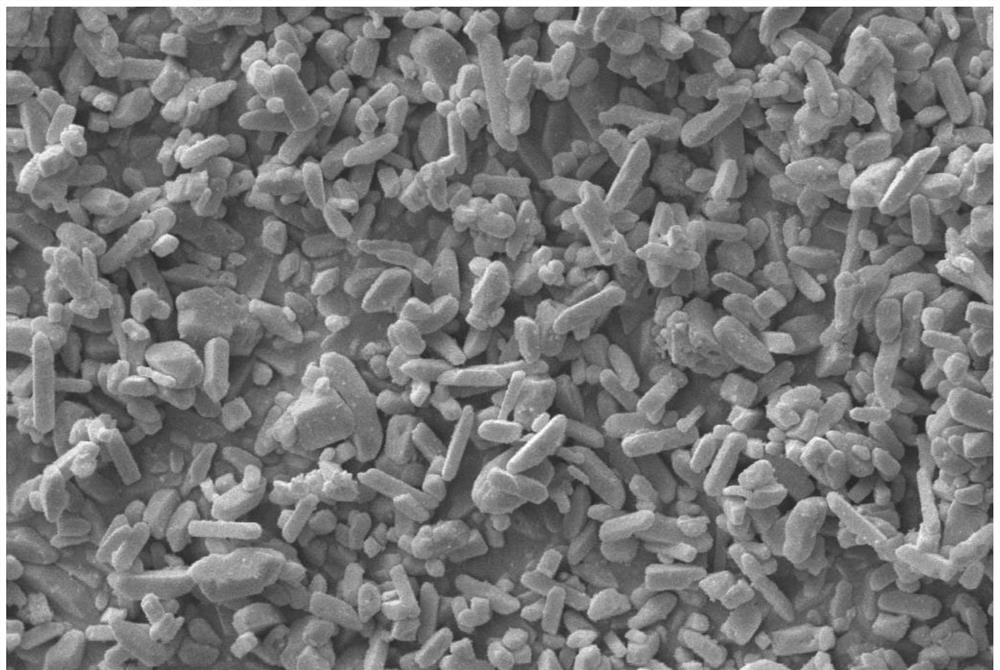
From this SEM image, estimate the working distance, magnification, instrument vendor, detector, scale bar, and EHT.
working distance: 8.5 mm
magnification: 5 K X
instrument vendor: Zeiss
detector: SE1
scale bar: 1000 nm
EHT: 10 kV